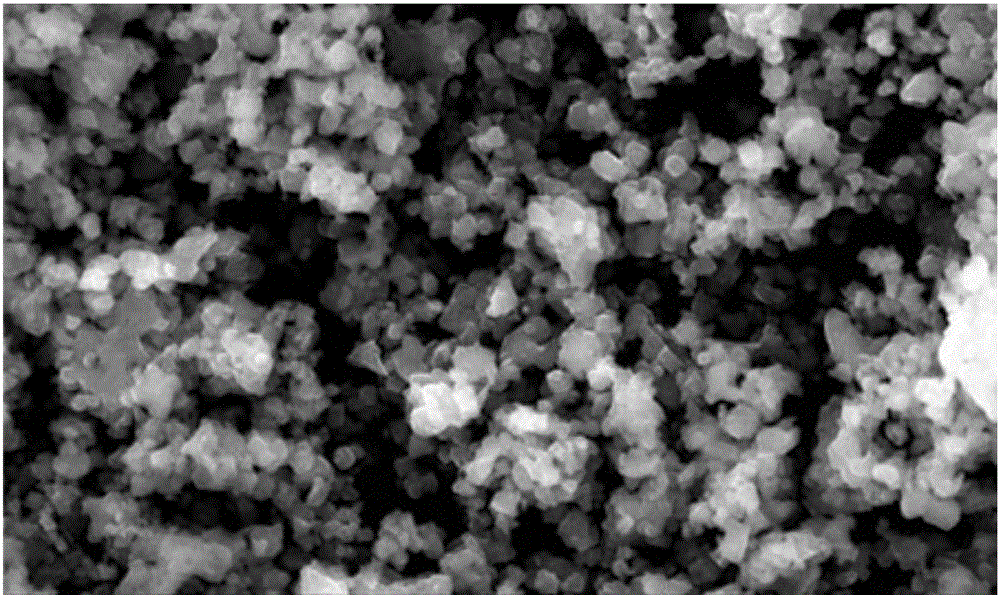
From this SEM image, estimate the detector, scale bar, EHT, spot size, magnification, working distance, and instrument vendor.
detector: ETD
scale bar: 5000 nm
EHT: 30 kV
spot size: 2.5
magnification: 10 K X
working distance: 10.3 mm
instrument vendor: FEI